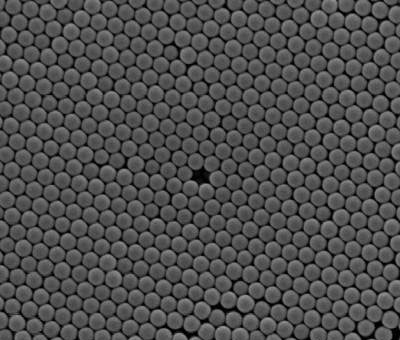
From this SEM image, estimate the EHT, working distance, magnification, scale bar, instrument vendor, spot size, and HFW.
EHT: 10 kV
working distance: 9.9 mm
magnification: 25 K X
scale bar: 2000 nm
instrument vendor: FEI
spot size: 3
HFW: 5.12 µm